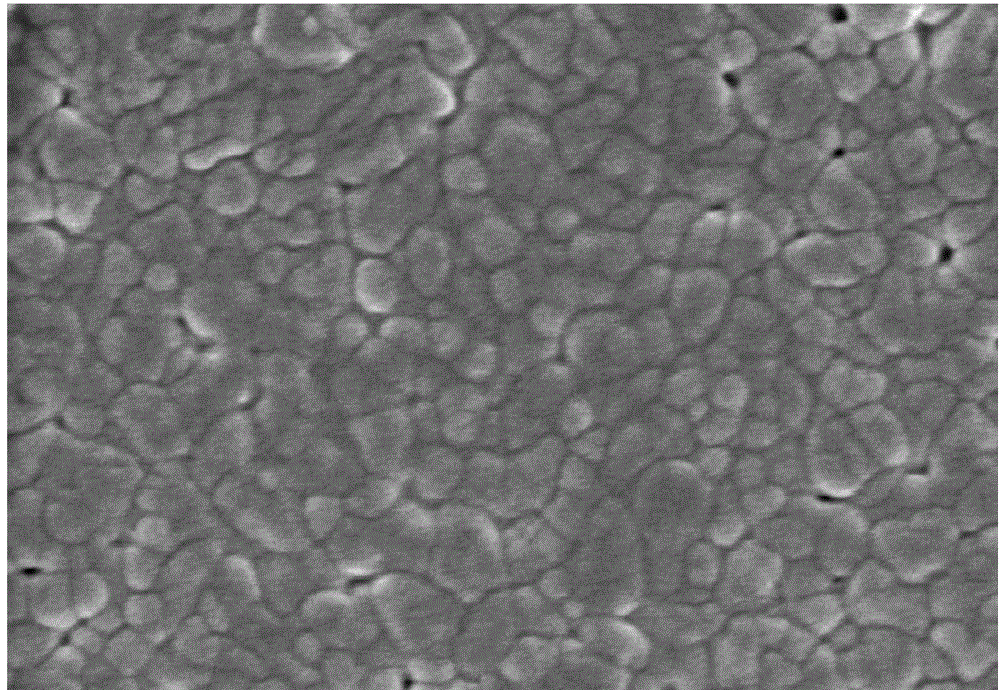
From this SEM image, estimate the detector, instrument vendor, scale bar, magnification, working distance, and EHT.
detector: SE(M)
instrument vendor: Hitachi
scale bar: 500 nm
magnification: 70 K X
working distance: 5.8 mm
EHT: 3 kV